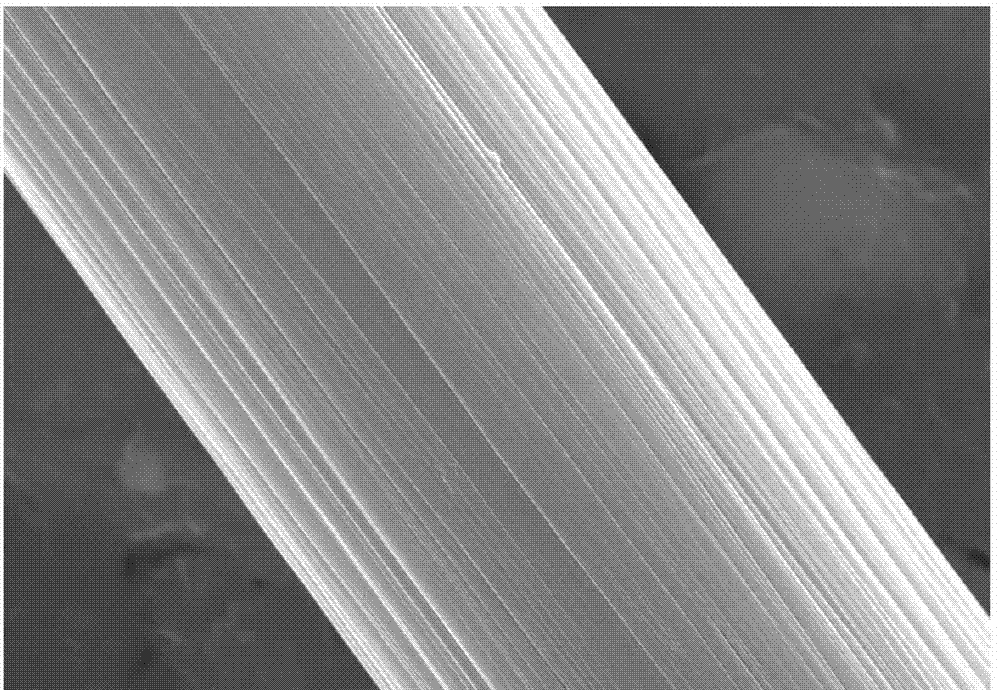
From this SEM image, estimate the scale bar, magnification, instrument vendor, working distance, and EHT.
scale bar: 5000 nm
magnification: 10 K X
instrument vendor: Hitachi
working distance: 14.8 mm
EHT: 20 kV